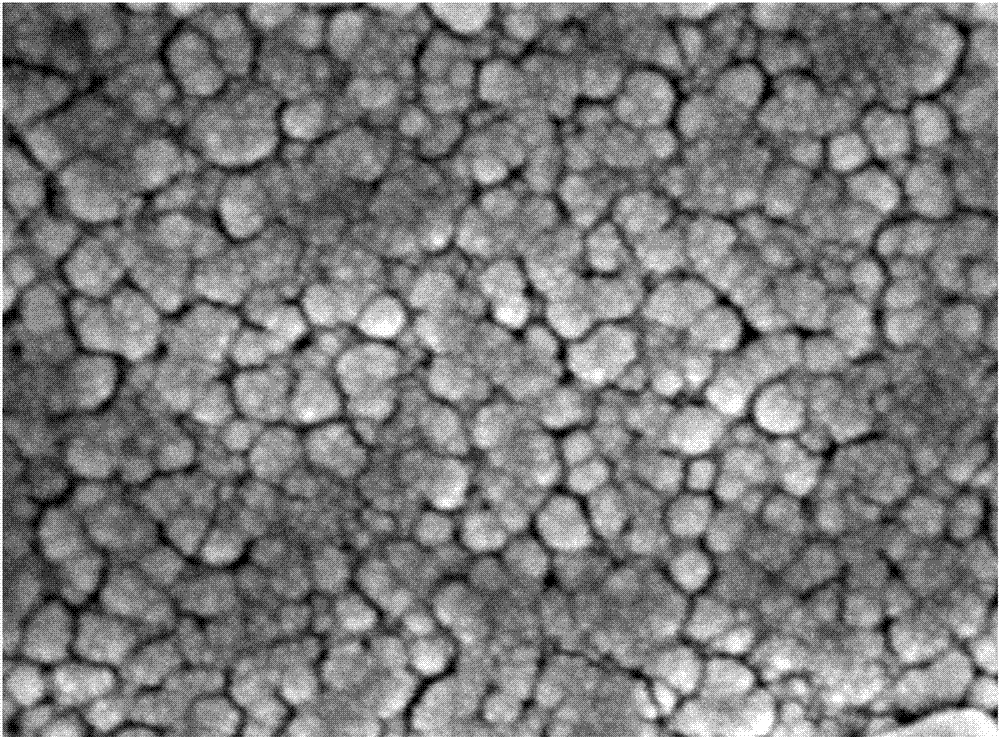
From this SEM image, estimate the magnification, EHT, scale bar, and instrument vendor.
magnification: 100 K X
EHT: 5 kV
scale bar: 100 nm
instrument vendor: Hitachi